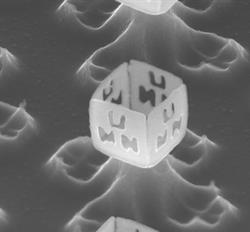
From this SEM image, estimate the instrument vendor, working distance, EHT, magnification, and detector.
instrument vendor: JEOL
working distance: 5.4 mm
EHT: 10 kV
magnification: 50 K X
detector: SEI